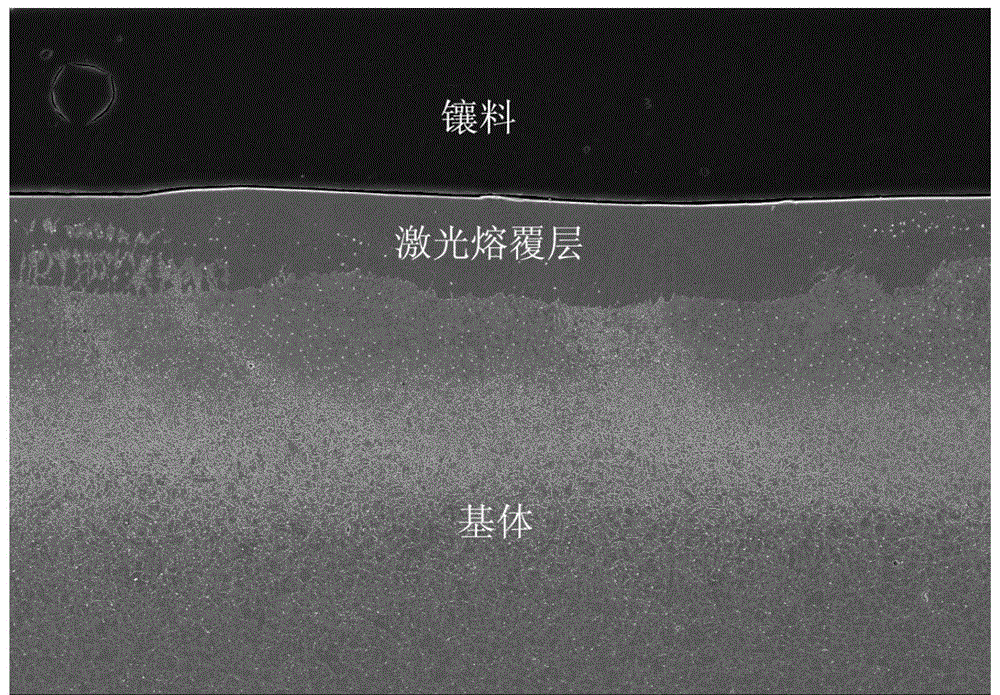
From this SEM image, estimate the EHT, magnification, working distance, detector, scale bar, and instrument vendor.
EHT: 20 kV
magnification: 0.2 K X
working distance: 11.1 mm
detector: SE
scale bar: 200000 nm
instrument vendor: Hitachi